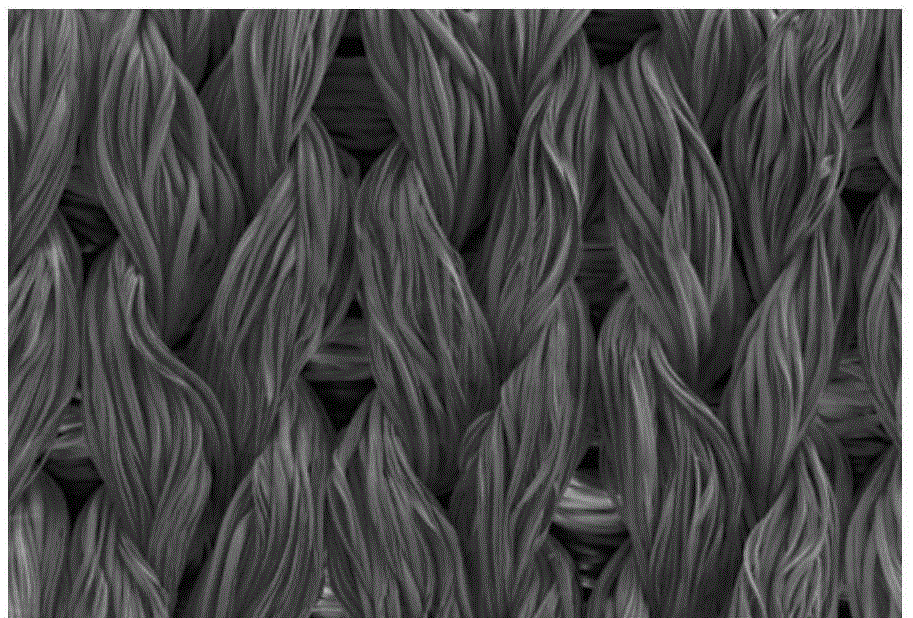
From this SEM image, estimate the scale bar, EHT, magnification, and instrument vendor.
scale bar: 100000 nm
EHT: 5 kV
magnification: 0.1 K X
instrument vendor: other